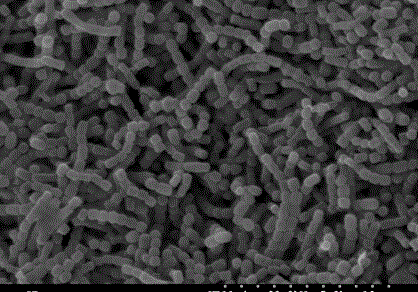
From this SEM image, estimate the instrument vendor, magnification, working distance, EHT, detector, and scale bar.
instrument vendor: Hitachi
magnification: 5 K X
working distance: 11.4 mm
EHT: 20 kV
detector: SE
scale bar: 10000 nm